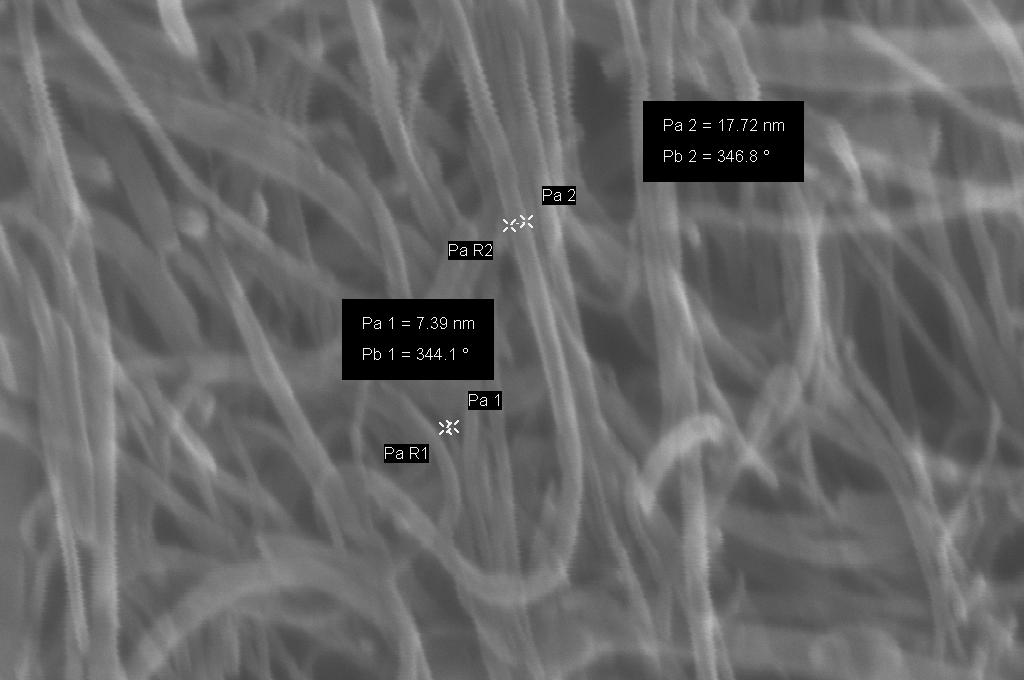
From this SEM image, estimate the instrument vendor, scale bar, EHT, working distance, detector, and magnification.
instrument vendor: Zeiss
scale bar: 100 nm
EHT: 20 kV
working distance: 4 mm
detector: InLens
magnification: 110.01 K X